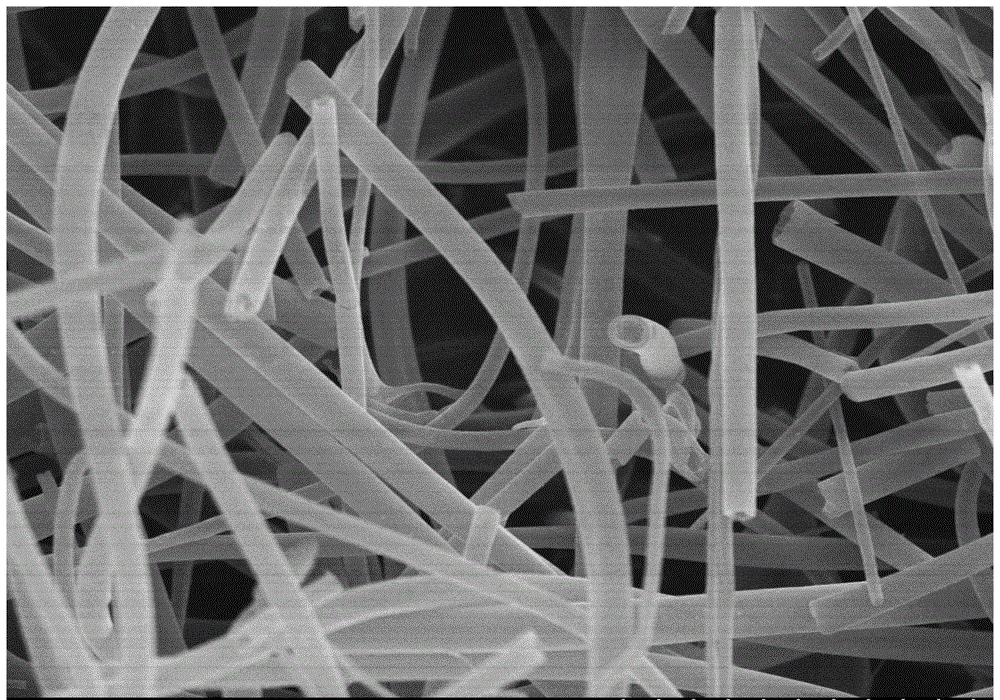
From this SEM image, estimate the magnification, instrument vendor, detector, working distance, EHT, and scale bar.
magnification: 9 K X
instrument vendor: Hitachi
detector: SE(U)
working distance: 10.3 mm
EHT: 5 kV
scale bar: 5000 nm